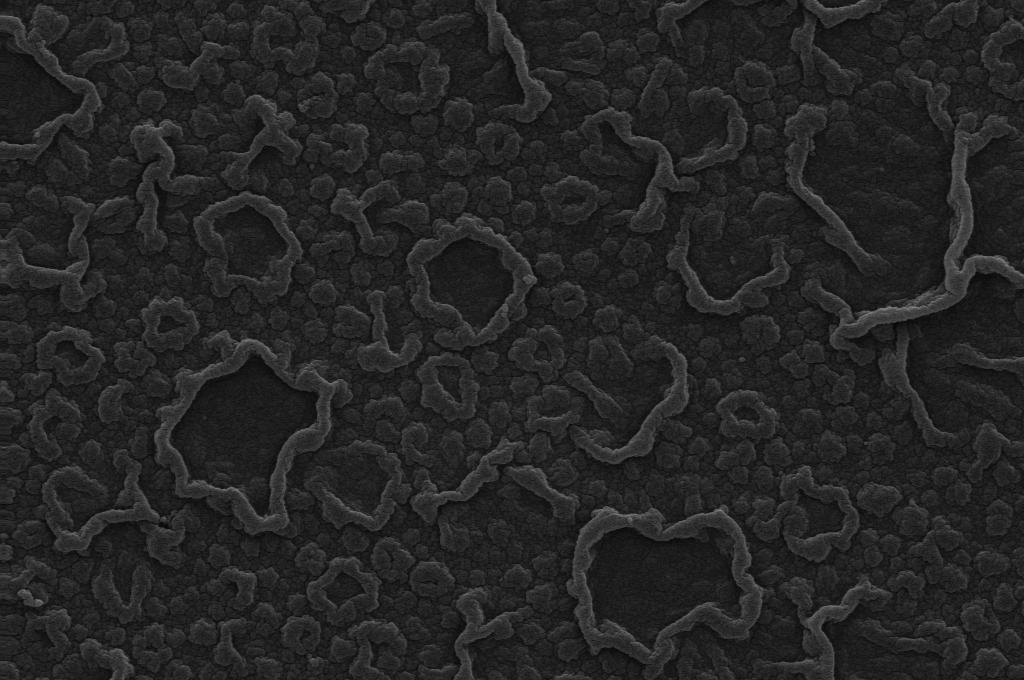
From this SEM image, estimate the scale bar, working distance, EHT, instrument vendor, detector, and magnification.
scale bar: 1000 nm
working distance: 9.5 mm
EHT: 20 kV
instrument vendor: Zeiss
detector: SE1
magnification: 5 K X